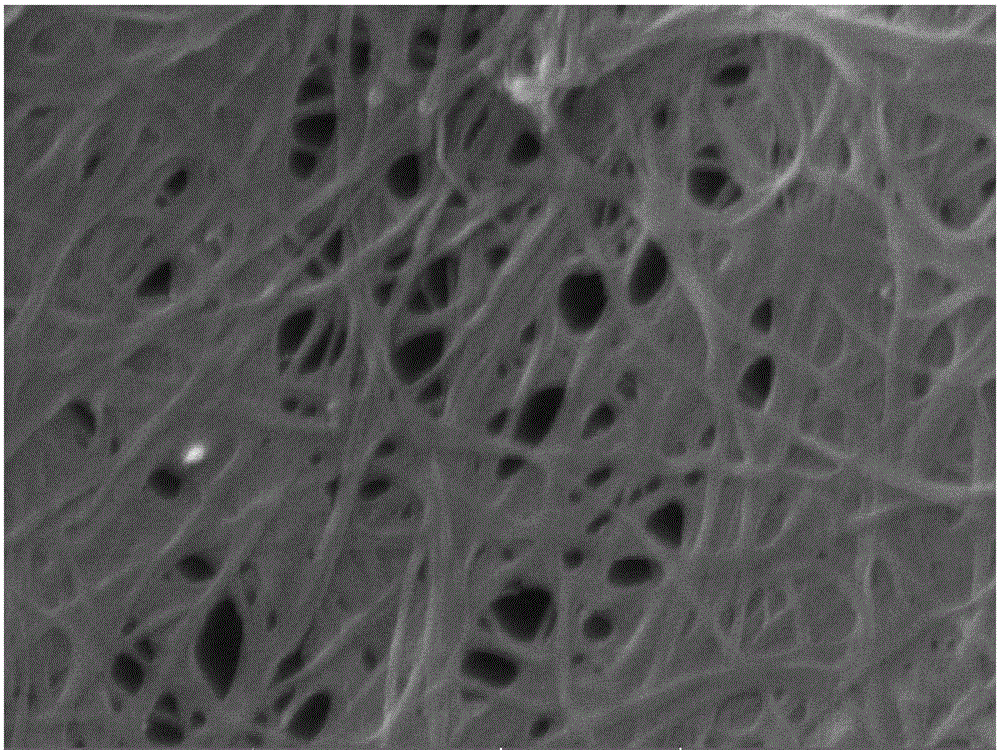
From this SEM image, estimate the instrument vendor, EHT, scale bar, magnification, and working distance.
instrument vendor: Tescan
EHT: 20 kV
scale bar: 500 nm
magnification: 100 K X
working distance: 15.58 mm